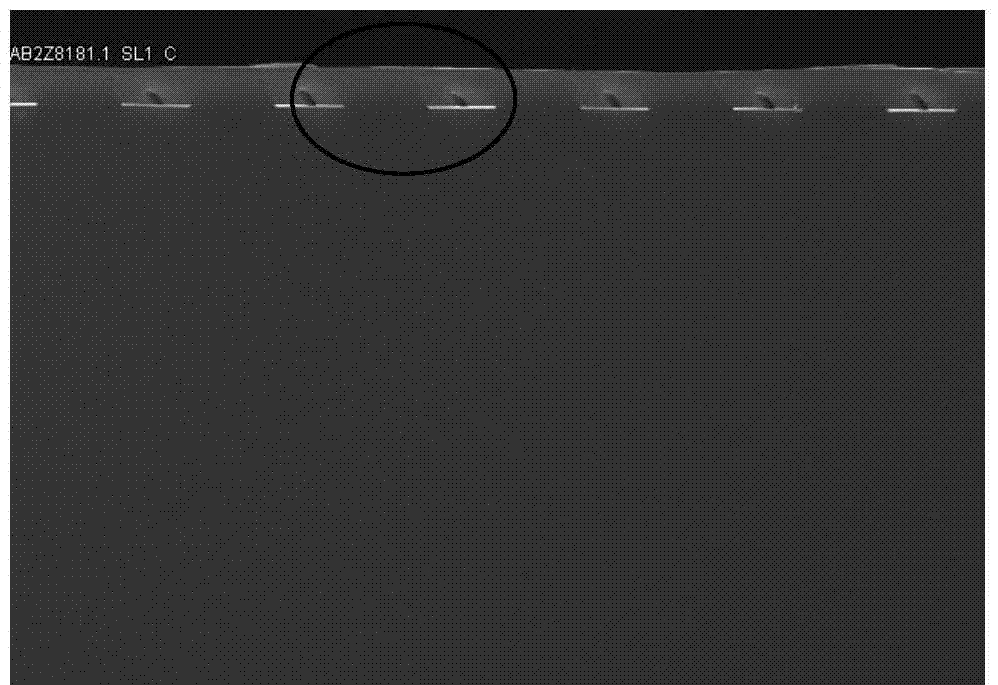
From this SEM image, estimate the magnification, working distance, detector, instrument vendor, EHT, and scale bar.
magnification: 2 K X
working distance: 9.7 mm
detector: SE(U)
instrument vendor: Hitachi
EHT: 15 kV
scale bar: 20000 nm